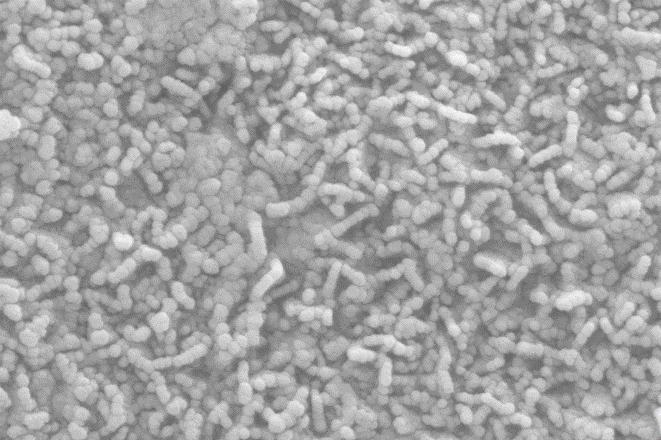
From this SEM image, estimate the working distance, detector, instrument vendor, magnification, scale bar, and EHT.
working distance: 8.3 mm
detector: SE2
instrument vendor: Zeiss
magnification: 100.71 K X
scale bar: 200 nm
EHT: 10 kV